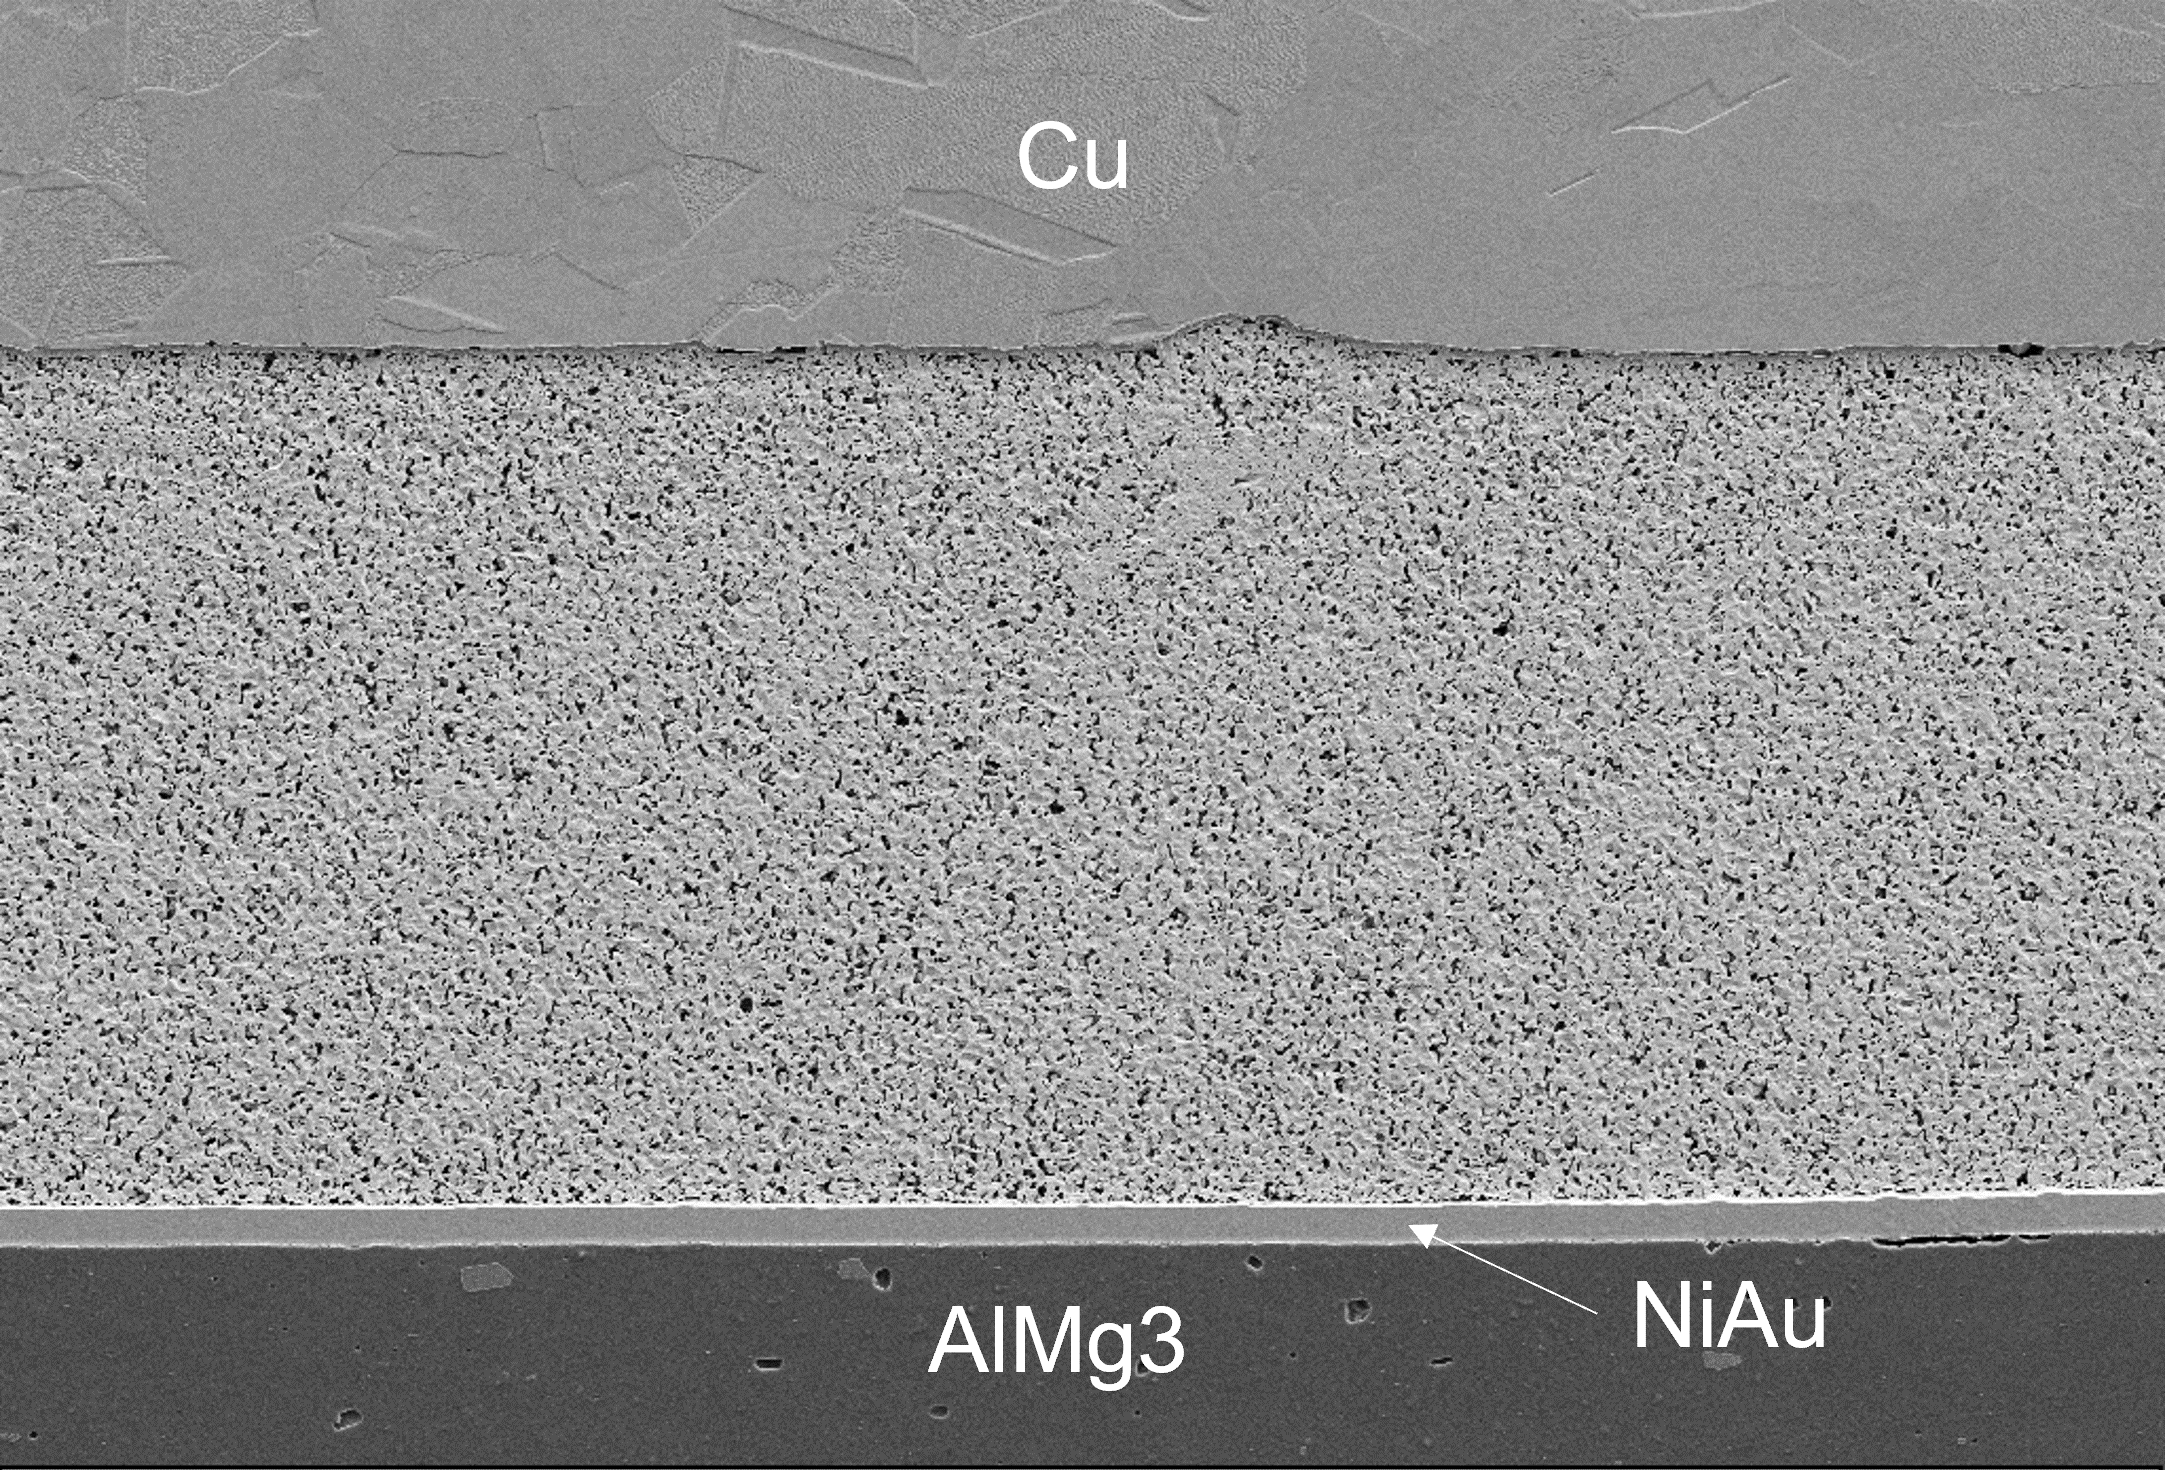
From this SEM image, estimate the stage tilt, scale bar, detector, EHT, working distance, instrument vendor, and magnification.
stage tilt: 0°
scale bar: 10000 nm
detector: SE2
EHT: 5 kV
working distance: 4.9 mm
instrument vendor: Zeiss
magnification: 1 K X